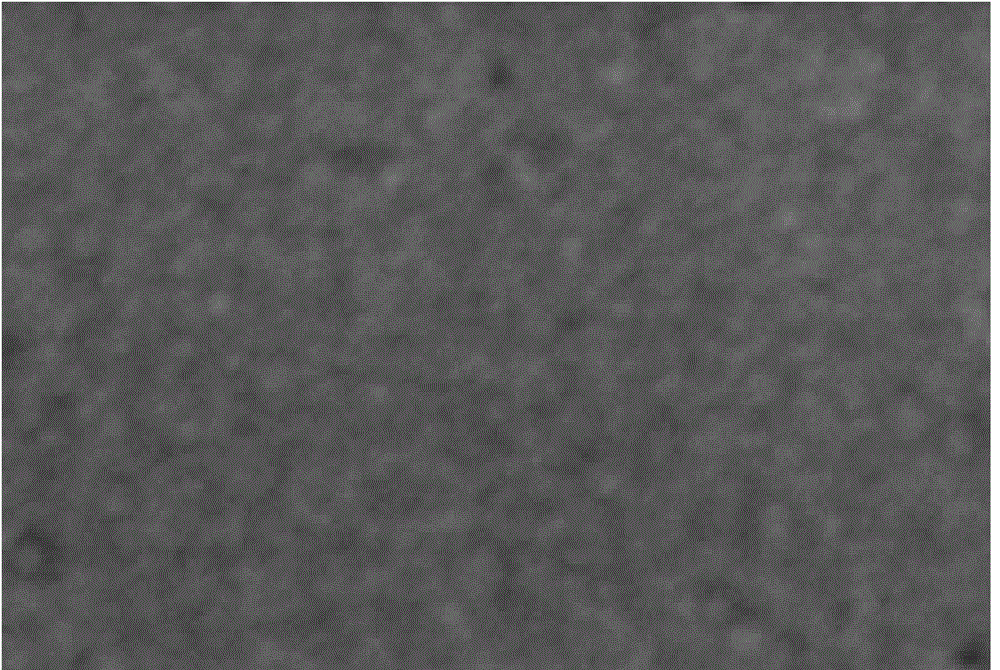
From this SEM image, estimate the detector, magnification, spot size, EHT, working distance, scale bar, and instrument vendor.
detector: SEI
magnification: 3 K X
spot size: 80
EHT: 30 kV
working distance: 14 mm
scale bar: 5000 nm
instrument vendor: JEOL